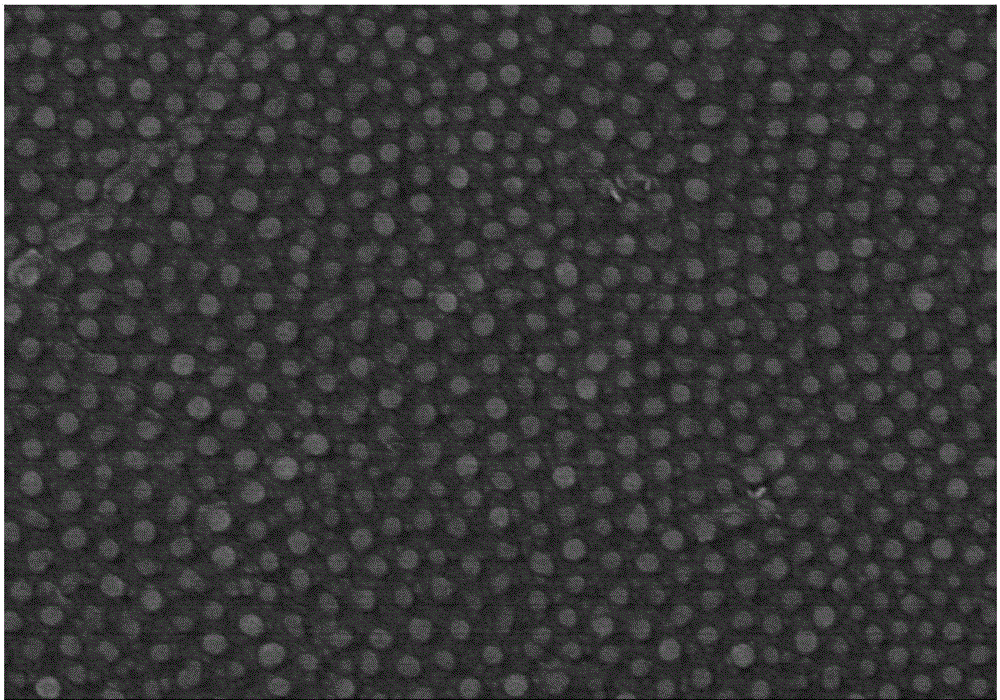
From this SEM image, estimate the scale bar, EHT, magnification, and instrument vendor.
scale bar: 1000 nm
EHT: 5 kV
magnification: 50 K X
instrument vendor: Hitachi